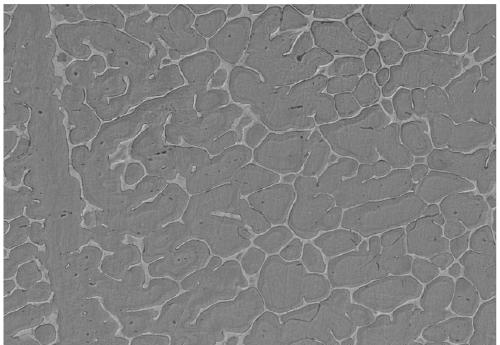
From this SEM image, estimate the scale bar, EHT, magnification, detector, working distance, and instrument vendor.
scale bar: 50000 nm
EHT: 15 kV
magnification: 1 K X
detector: SE(UL)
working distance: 9.2 mm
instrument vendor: Hitachi